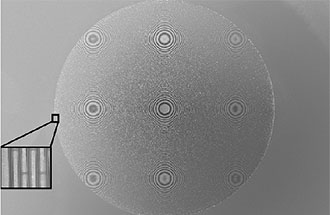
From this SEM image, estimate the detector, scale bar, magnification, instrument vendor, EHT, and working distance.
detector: InLens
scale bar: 20000 nm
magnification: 0.249 K X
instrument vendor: Zeiss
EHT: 15 kV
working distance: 8.1 mm